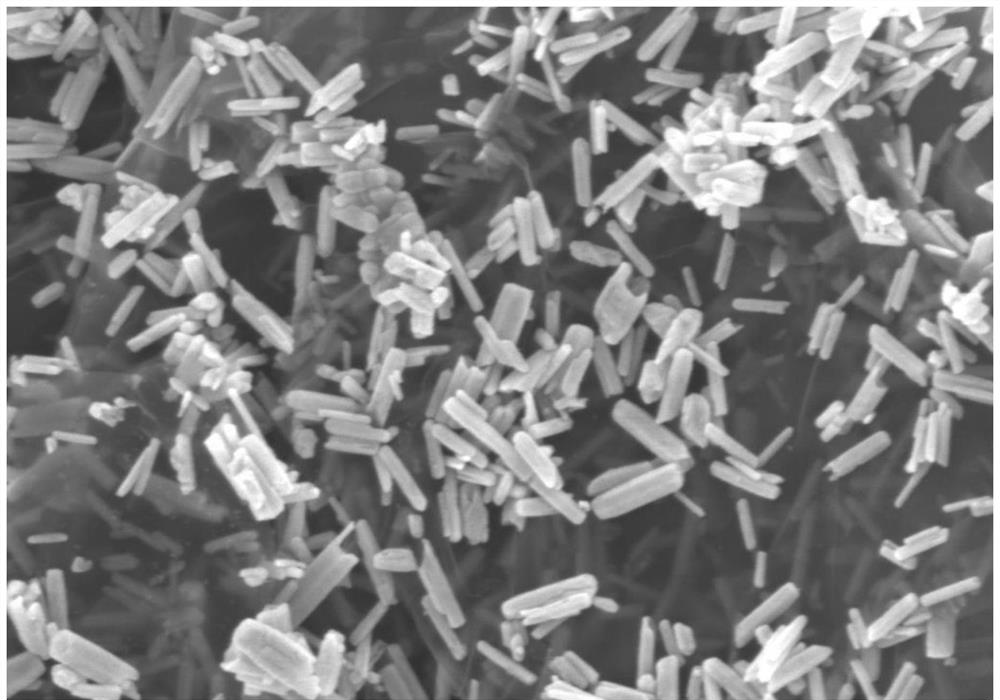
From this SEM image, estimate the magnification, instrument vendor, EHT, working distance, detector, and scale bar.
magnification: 43.17 K X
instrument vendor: Zeiss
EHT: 3 kV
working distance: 8.7 mm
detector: InLens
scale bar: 200 nm